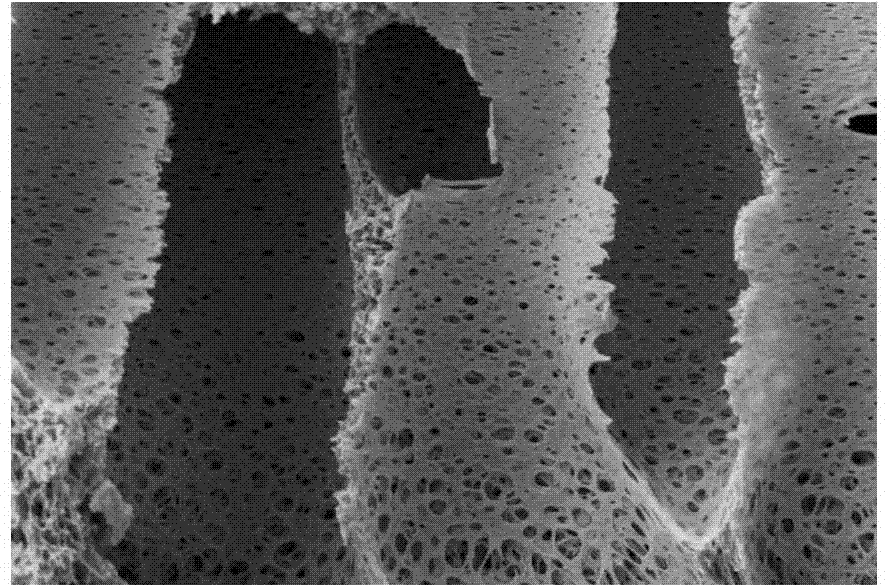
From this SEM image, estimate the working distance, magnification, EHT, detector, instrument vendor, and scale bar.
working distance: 5.8 mm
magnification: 3 K X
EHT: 6 kV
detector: SE2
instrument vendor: Zeiss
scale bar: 2000 nm